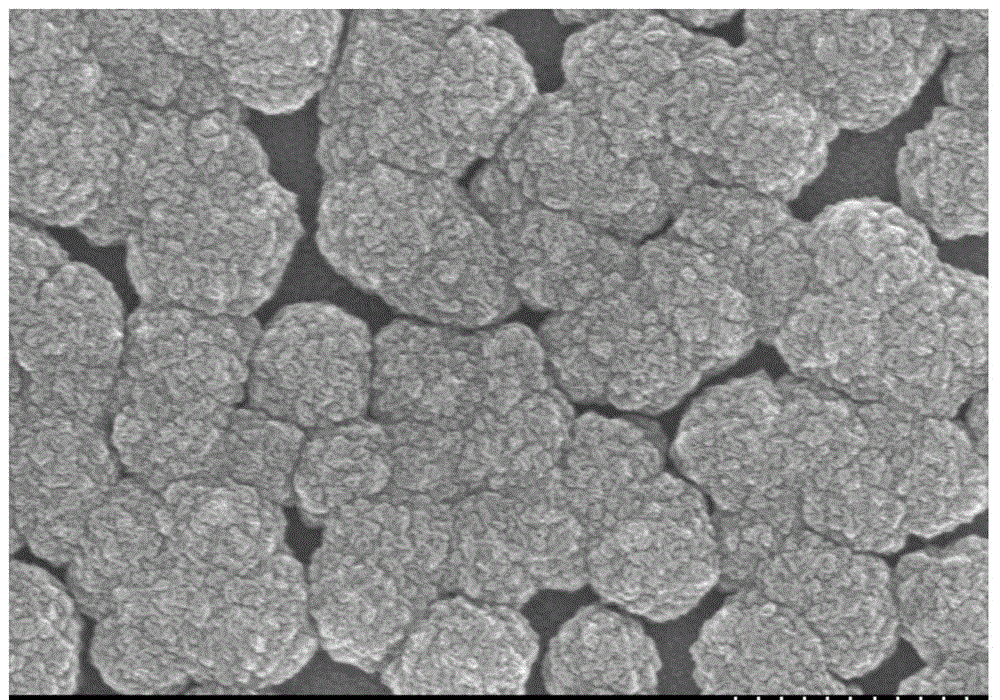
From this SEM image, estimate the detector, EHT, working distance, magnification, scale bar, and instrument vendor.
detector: SE(M)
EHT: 15 kV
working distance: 12.8 mm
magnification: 30 K X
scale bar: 1000 nm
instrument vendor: Hitachi